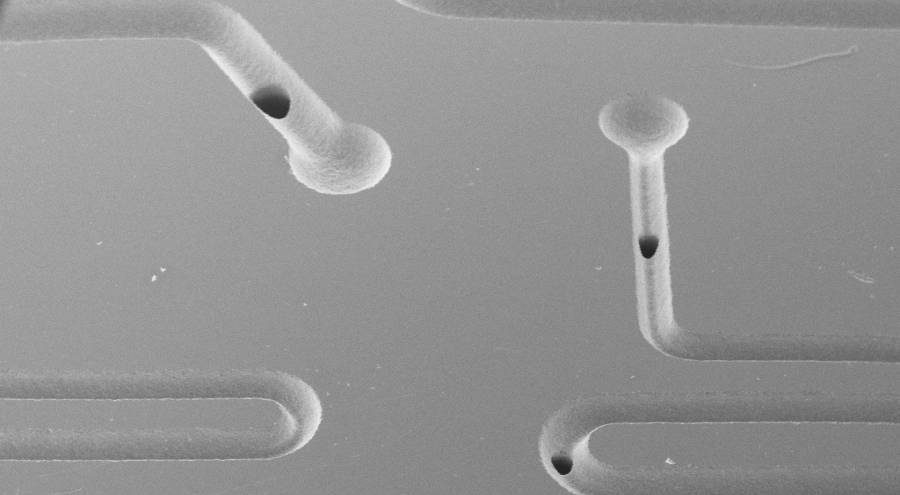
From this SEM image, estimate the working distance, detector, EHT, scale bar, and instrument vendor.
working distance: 21 mm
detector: SE1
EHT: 20 kV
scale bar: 1000000 nm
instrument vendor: Zeiss